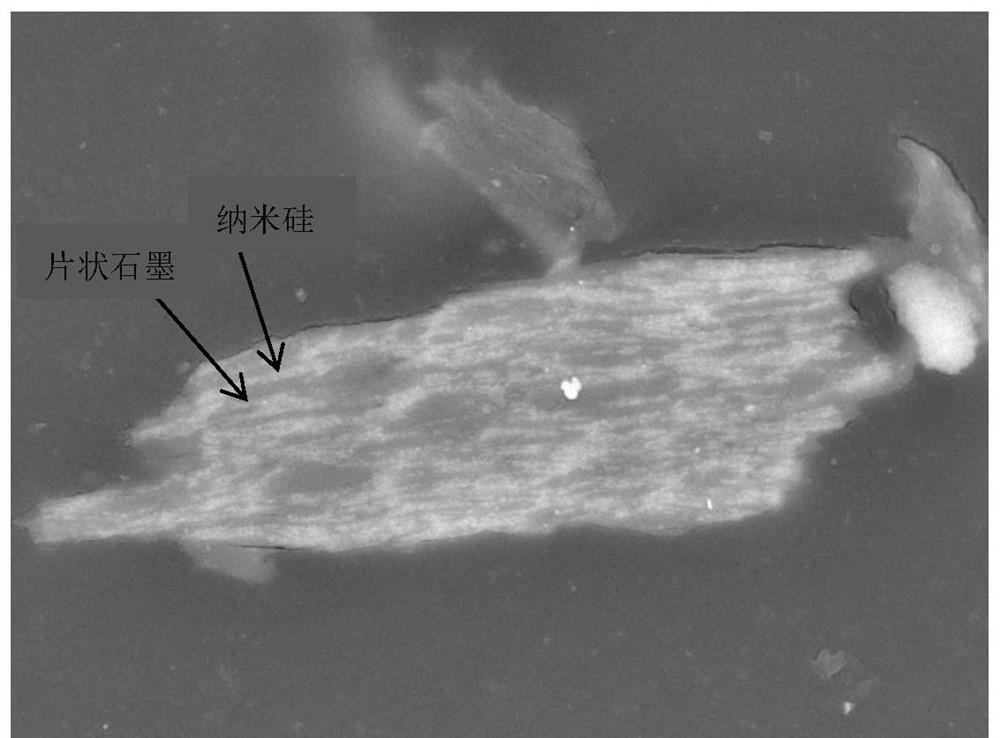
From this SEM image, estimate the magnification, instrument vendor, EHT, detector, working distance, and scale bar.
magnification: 5 K X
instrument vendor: JEOL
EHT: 15 kV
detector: BED-C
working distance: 8.3 mm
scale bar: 1000 nm